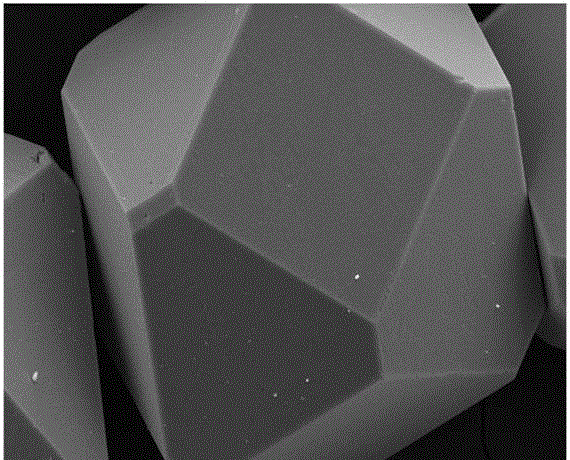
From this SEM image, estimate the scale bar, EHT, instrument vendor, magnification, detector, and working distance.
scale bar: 10000 nm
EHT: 20 kV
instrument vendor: Zeiss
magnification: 0.8 K X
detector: SE2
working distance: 7.2 mm